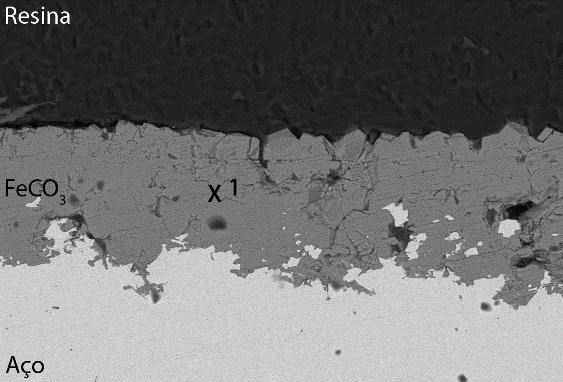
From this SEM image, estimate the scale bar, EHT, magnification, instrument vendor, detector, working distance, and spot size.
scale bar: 50000 nm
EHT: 15 kV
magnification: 0.5 K X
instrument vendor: JEOL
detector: BEC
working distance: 11 mm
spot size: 40